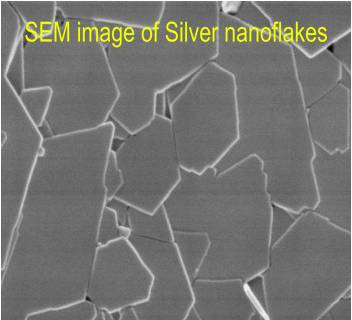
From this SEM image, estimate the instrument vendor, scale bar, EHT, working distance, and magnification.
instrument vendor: Hitachi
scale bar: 1000 nm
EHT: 1 kV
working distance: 9.1 mm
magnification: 50 K X